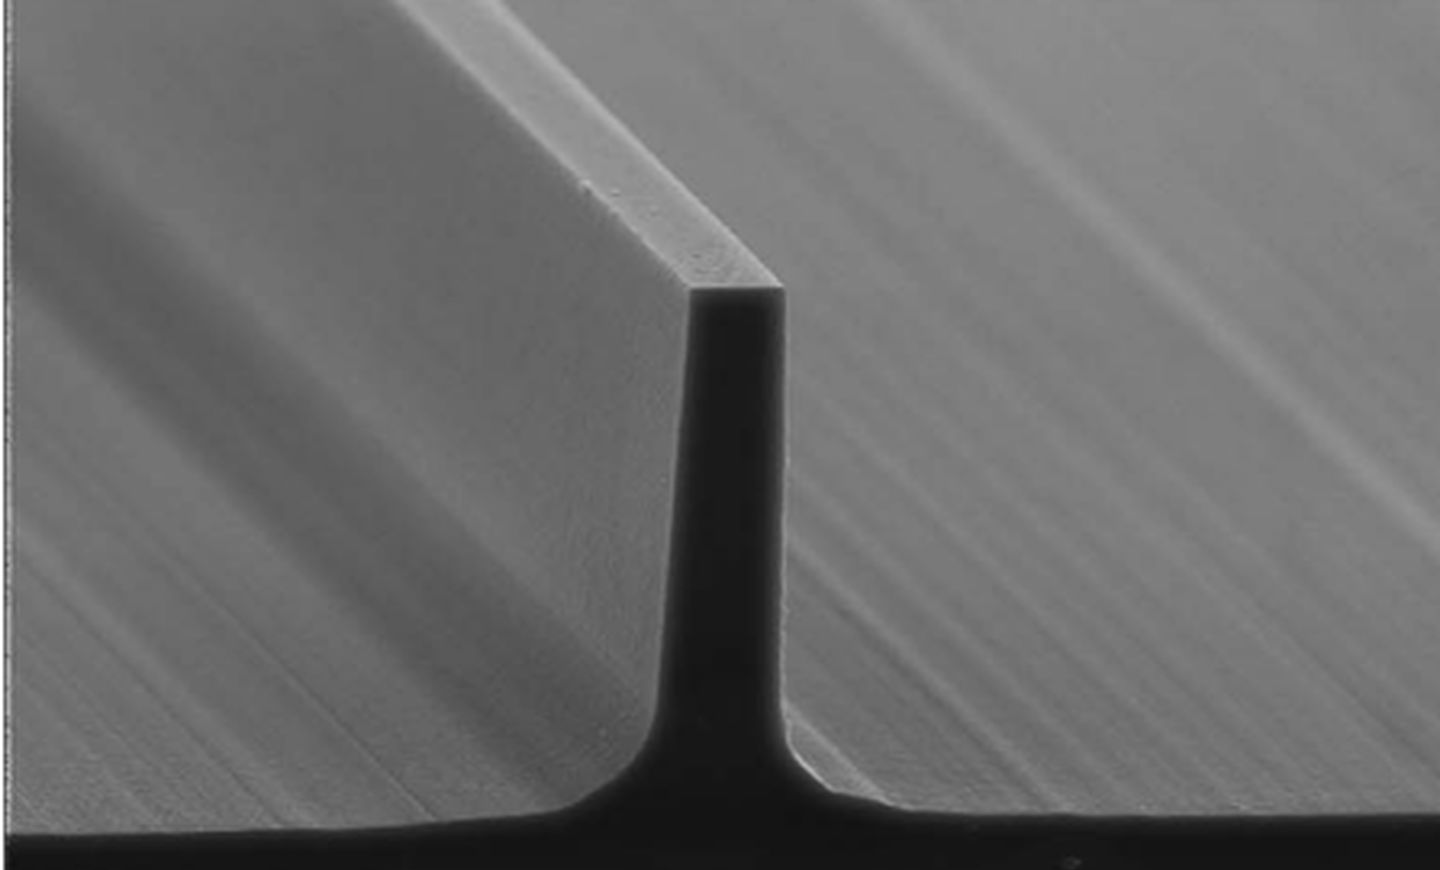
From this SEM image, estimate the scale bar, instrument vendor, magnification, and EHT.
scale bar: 20000 nm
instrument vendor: Hitachi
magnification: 1.5 K X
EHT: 20 kV